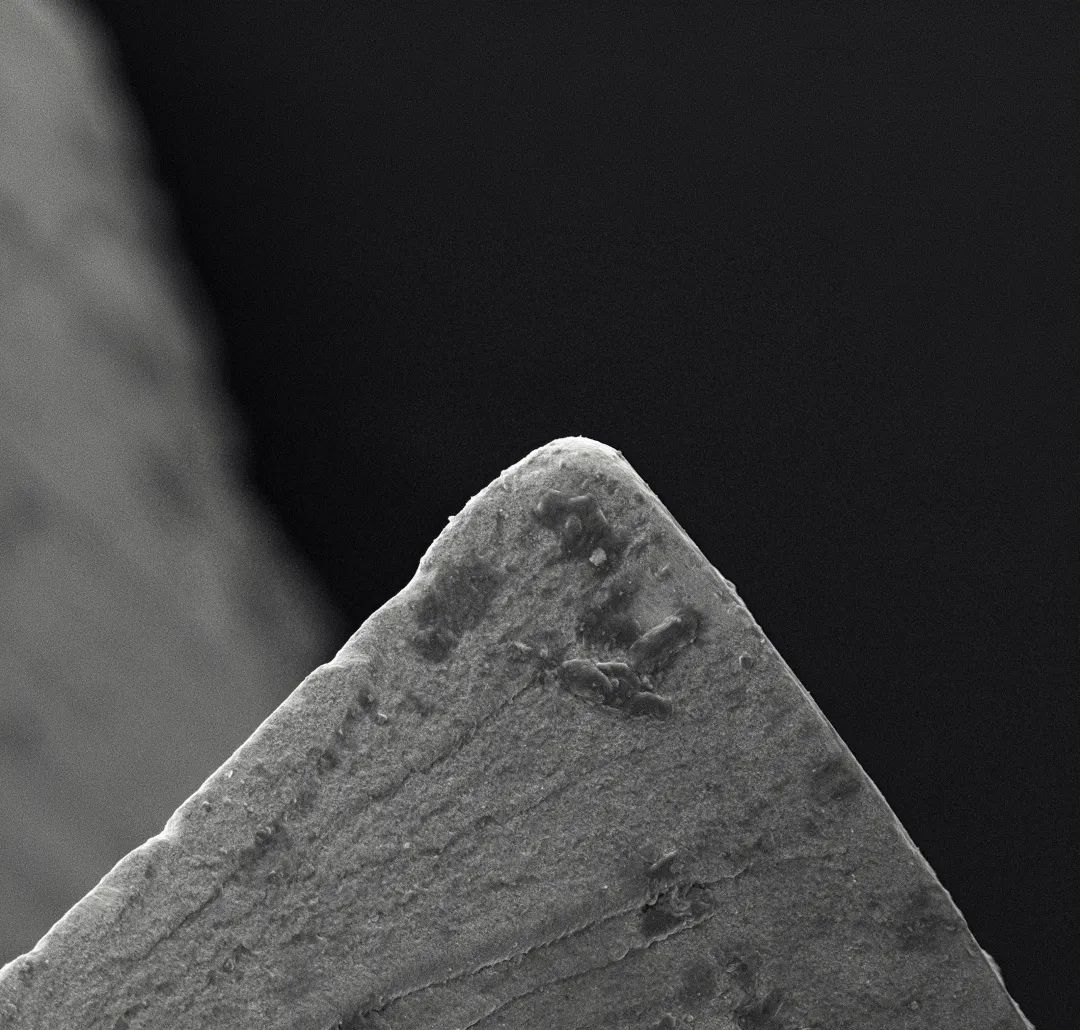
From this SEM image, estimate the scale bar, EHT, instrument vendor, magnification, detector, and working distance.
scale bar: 100000 nm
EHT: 15 kV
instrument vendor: other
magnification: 0.5 K X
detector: SE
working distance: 8.9 mm